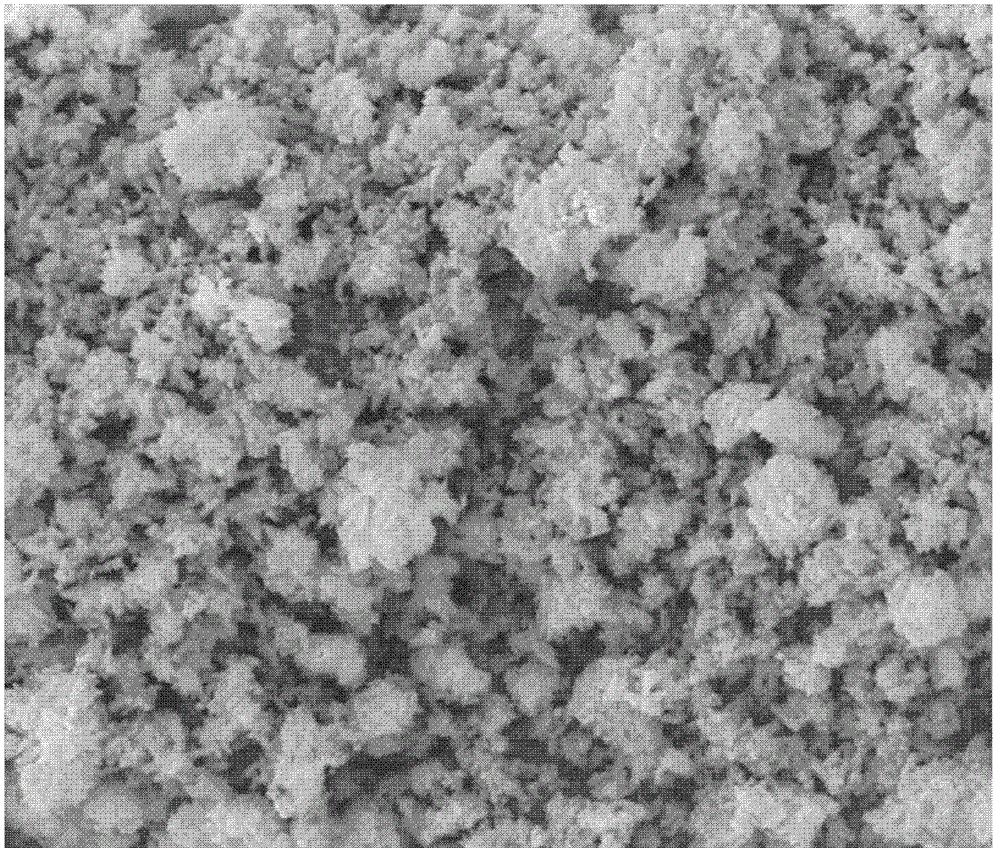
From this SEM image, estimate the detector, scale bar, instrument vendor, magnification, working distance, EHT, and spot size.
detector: ETD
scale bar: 10000 nm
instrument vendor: FEI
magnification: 3 K X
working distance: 10.1 mm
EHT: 15 kV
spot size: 4.5